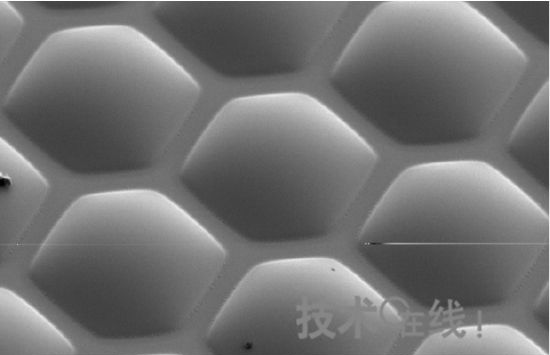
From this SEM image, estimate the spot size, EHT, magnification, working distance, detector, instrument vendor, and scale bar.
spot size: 3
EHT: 3 kV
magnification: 0.5 K X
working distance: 4.8 mm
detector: SE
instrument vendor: FEI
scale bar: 50000 nm